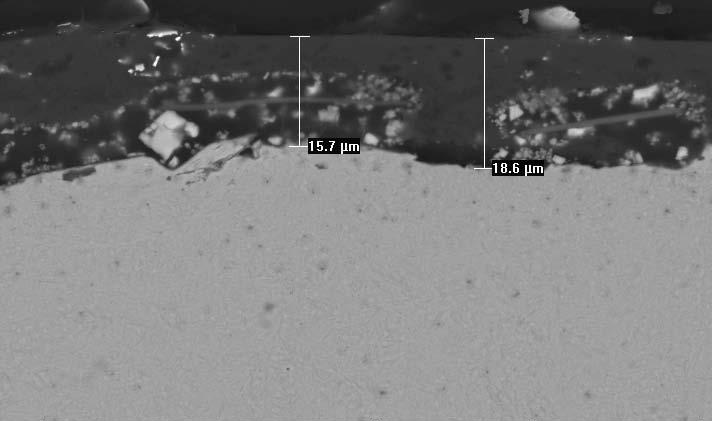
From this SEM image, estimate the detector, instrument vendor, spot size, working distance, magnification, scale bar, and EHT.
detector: BSE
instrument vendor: FEI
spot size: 4.2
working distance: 13.7 mm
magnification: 1 K X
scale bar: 20000 nm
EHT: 20 kV